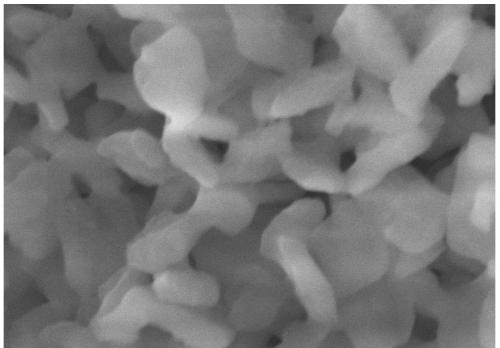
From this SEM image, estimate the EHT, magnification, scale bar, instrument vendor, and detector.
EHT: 5 kV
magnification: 100 K X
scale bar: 500 nm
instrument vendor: Hitachi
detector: SE(M)